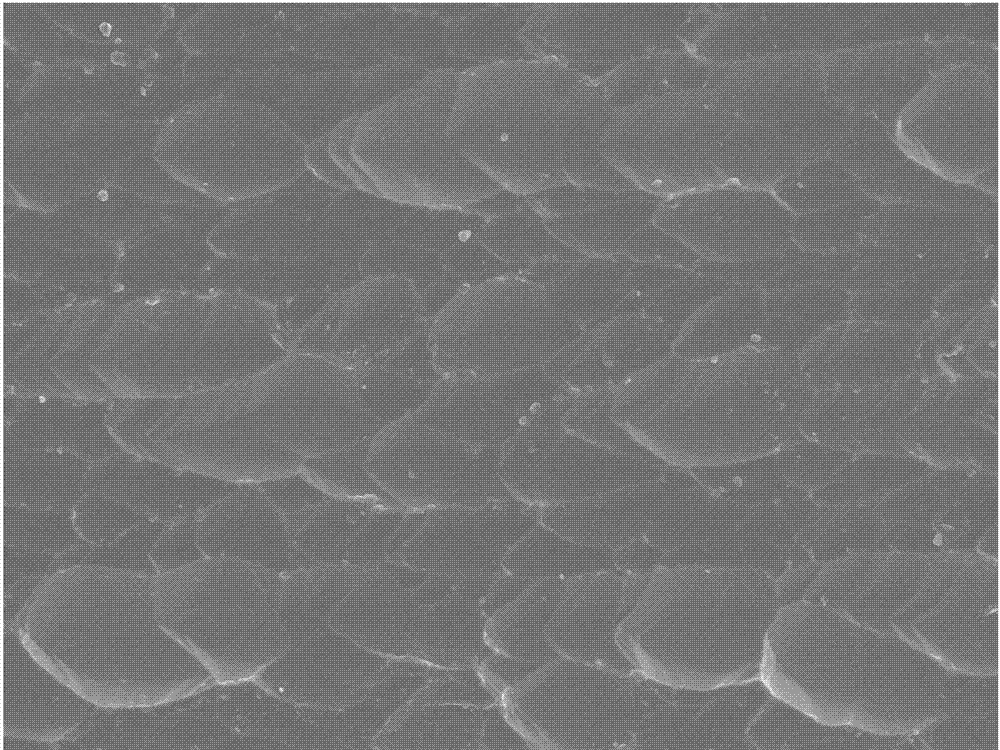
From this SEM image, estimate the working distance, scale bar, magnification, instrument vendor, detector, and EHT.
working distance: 8.2 mm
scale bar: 10000 nm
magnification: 2.5 K X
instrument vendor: JEOL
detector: SEI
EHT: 5 kV